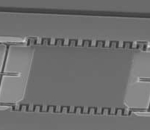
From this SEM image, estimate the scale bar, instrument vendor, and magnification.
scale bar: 20000 nm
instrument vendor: JEOL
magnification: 0.7 K X